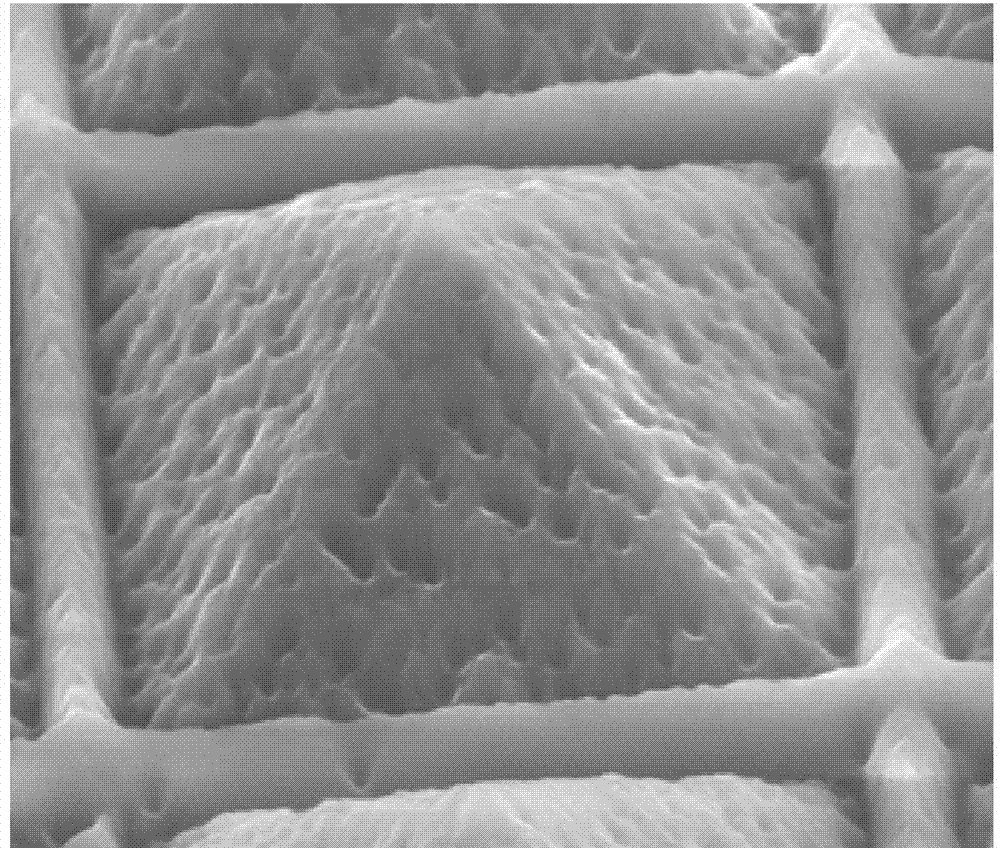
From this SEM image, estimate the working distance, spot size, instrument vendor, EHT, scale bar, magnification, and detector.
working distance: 15.2 mm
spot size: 3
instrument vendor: FEI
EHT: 10 kV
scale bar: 5000 nm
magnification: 15 K X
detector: LFD